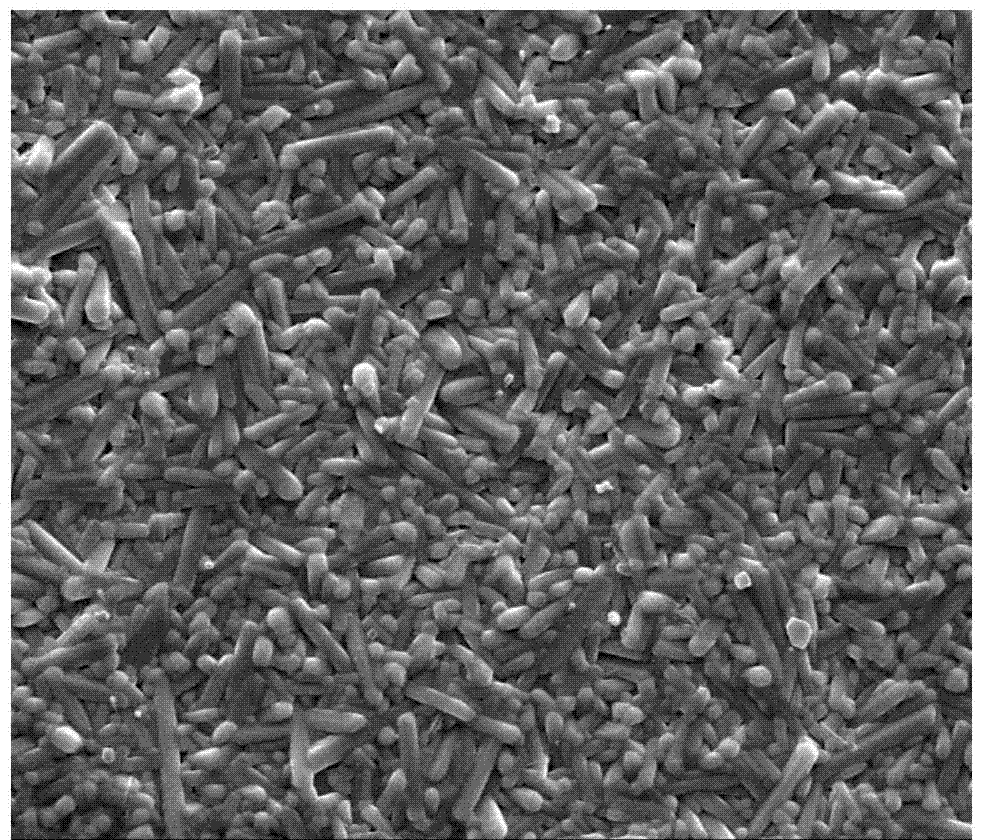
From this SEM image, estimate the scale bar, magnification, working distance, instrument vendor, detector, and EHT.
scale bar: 20000 nm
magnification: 5 K X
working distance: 11.2 mm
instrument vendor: FEI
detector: SE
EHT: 20 kV